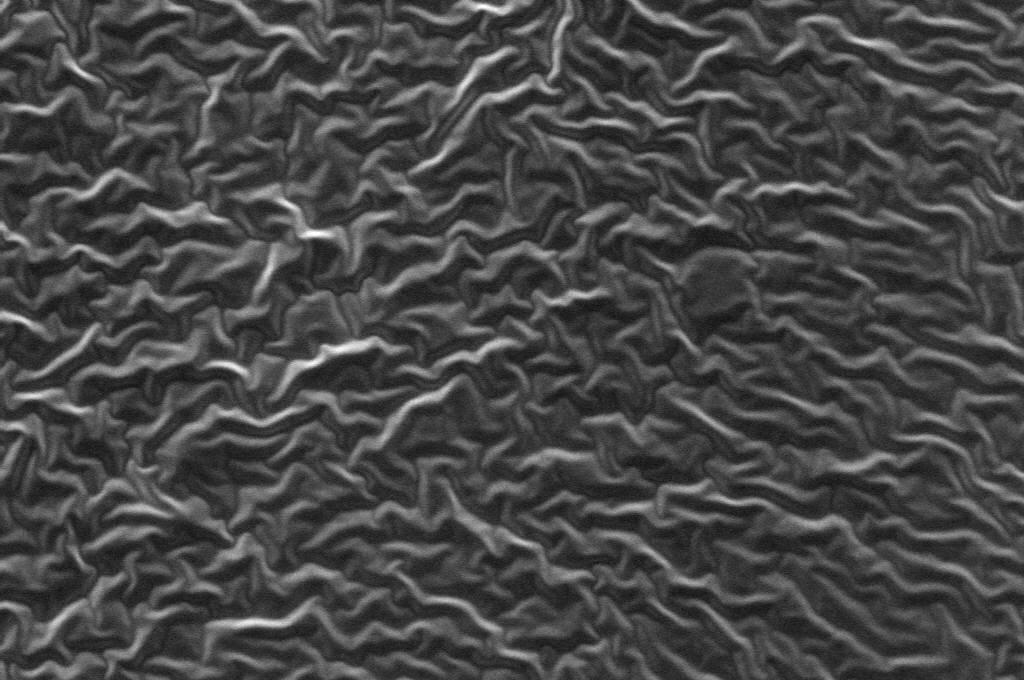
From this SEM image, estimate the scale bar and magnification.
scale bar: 50000 nm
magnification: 0.5 K X